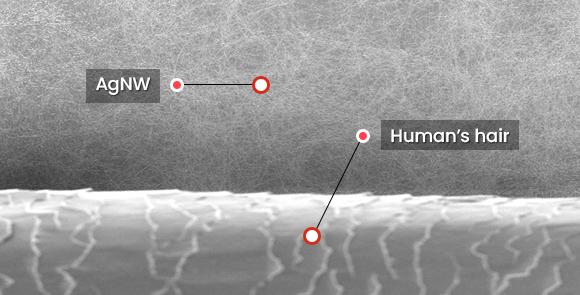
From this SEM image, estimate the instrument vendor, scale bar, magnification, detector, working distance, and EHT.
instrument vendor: Hitachi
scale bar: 50000 nm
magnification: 1 K X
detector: SE(UL)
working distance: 5.1 mm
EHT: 5 kV